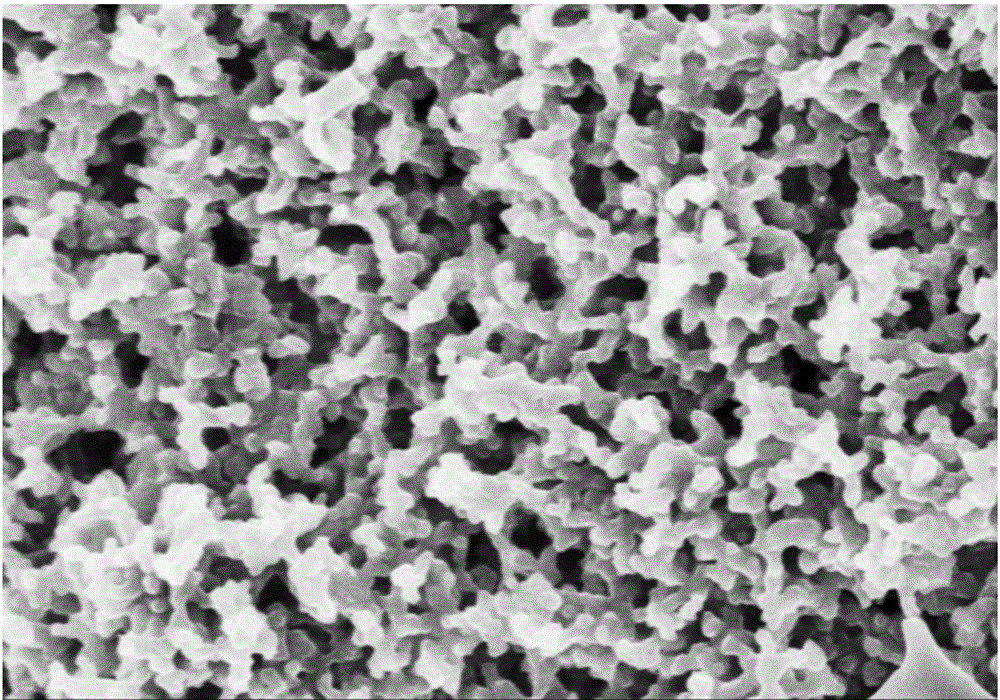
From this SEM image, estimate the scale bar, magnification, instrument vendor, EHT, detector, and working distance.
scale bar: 1000 nm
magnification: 30 K X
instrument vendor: Hitachi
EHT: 3 kV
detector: SE(M)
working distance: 7.7 mm